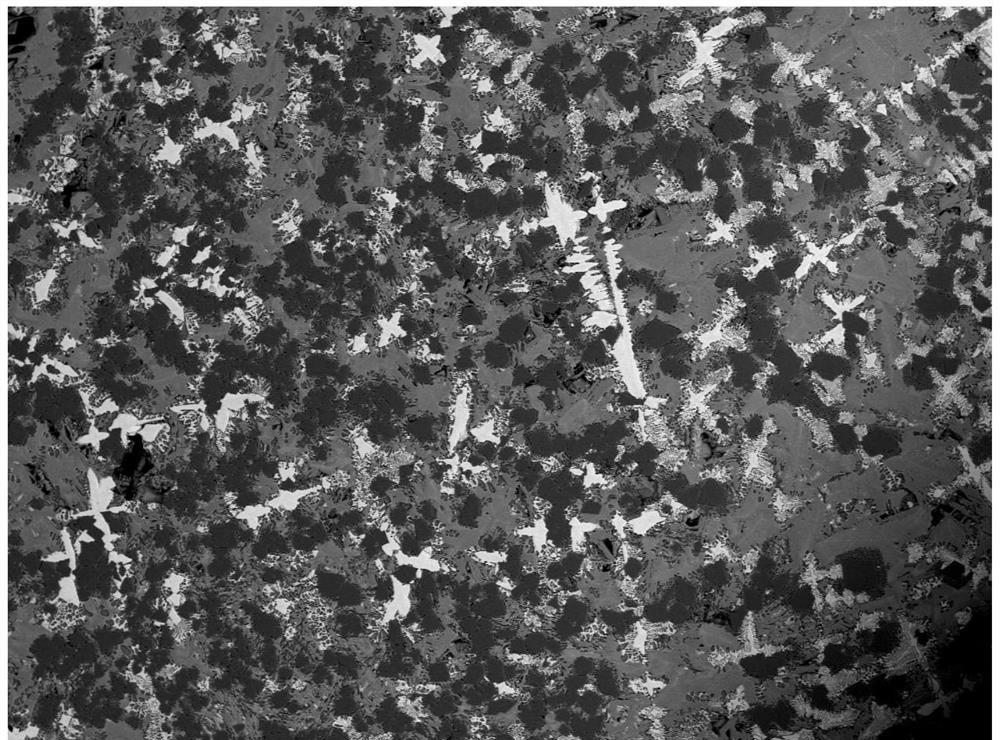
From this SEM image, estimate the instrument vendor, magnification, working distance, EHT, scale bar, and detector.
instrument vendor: JEOL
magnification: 0.05 K X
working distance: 9.4 mm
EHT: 20 kV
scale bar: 500000 nm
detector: BED-S